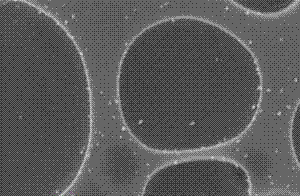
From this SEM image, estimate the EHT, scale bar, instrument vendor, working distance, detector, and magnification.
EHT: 10 kV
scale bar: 200 nm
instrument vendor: Zeiss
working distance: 7 mm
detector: InLens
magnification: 60 K X